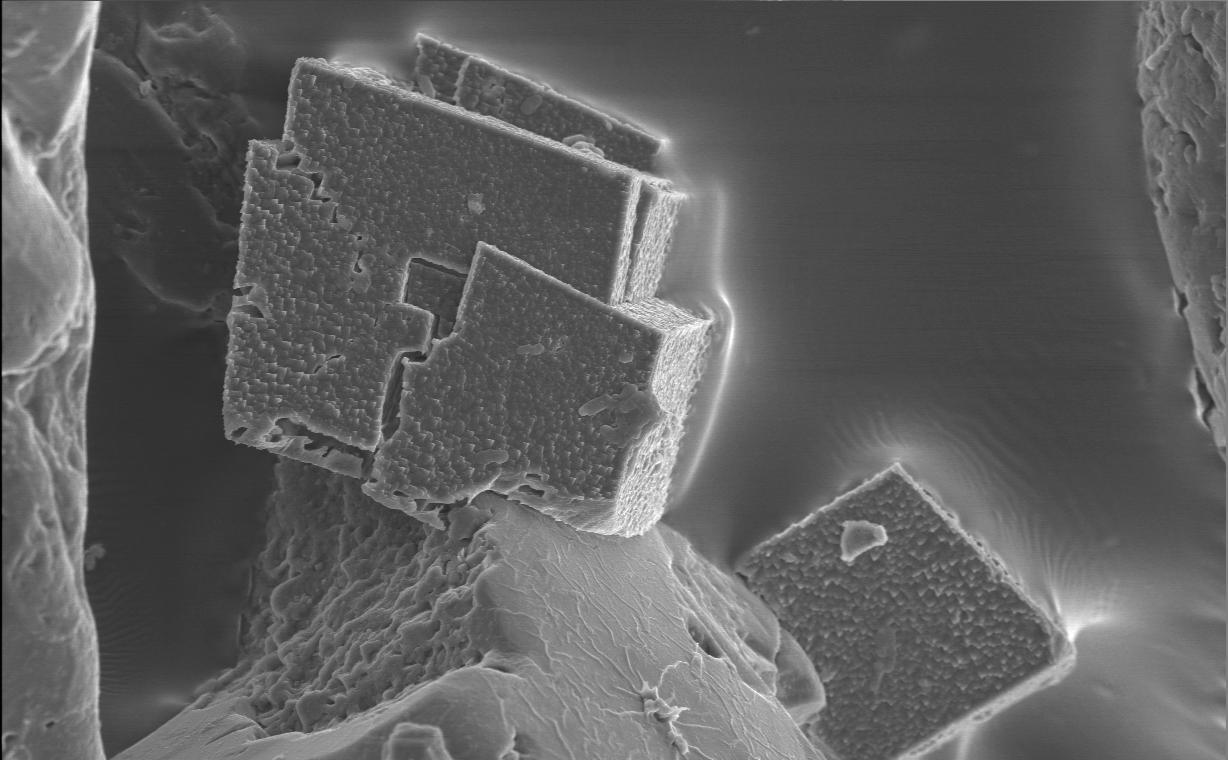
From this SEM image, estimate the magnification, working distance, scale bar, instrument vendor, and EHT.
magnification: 1.5 K X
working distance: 15 mm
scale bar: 10000 nm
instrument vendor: JEOL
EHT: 8 kV